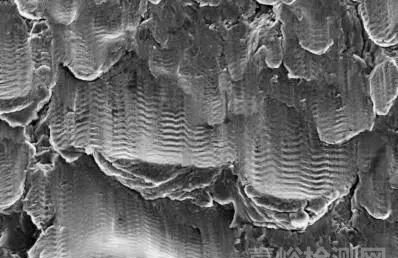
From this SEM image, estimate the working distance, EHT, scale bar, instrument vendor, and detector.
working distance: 29 mm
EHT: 20 kV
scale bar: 20000 nm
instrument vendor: Zeiss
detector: SE2